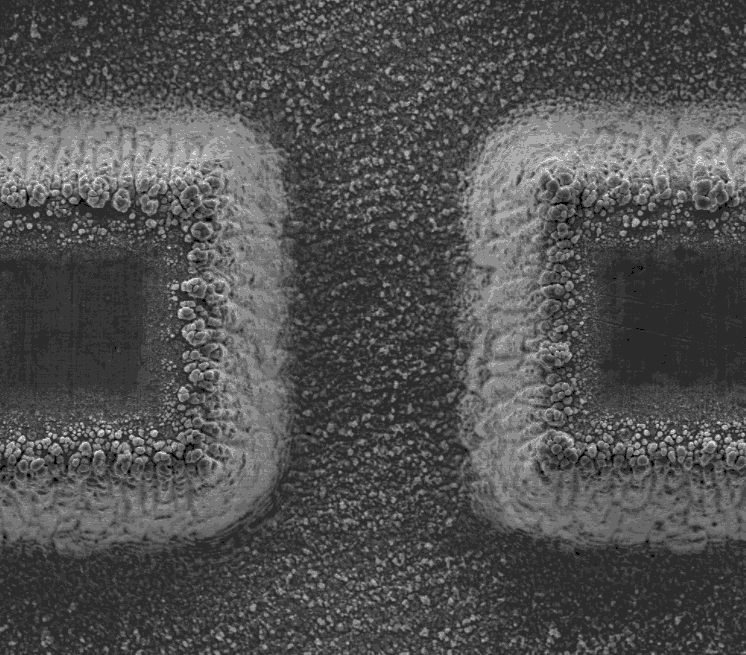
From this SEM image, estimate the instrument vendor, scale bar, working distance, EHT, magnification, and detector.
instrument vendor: FEI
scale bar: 100000 nm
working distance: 10.1 mm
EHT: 20 kV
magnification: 0.5 K X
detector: ETD